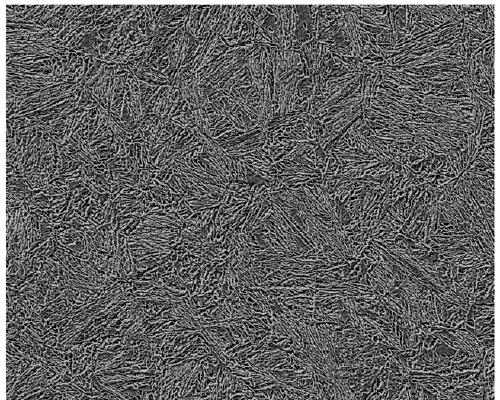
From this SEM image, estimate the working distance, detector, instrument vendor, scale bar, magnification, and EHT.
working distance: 8 mm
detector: SEI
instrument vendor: JEOL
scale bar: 10000 nm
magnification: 1 K X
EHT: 5 kV